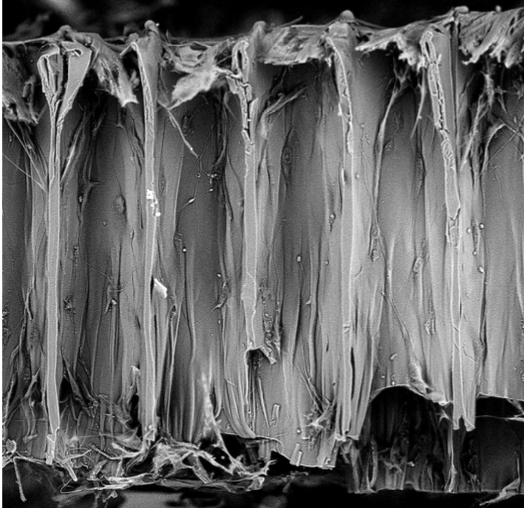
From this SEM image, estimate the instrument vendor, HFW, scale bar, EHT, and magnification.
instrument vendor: other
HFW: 484 µm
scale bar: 100000 nm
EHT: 10 kV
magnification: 0.56 K X